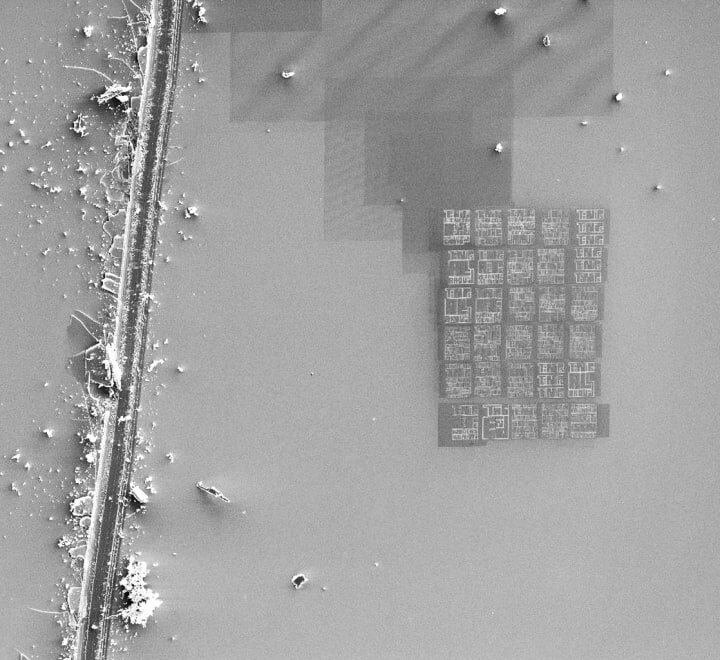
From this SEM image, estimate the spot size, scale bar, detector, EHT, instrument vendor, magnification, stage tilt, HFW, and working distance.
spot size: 3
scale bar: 50000 nm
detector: SED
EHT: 5 kV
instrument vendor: FEI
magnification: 0.5 K X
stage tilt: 0°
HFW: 304 µm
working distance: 5.01 mm